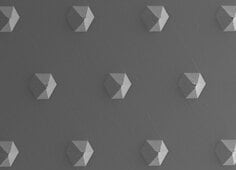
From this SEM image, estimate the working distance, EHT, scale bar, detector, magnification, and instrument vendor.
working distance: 11.7 mm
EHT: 15 kV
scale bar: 100000 nm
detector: SEI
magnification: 0.05 K X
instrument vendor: JEOL